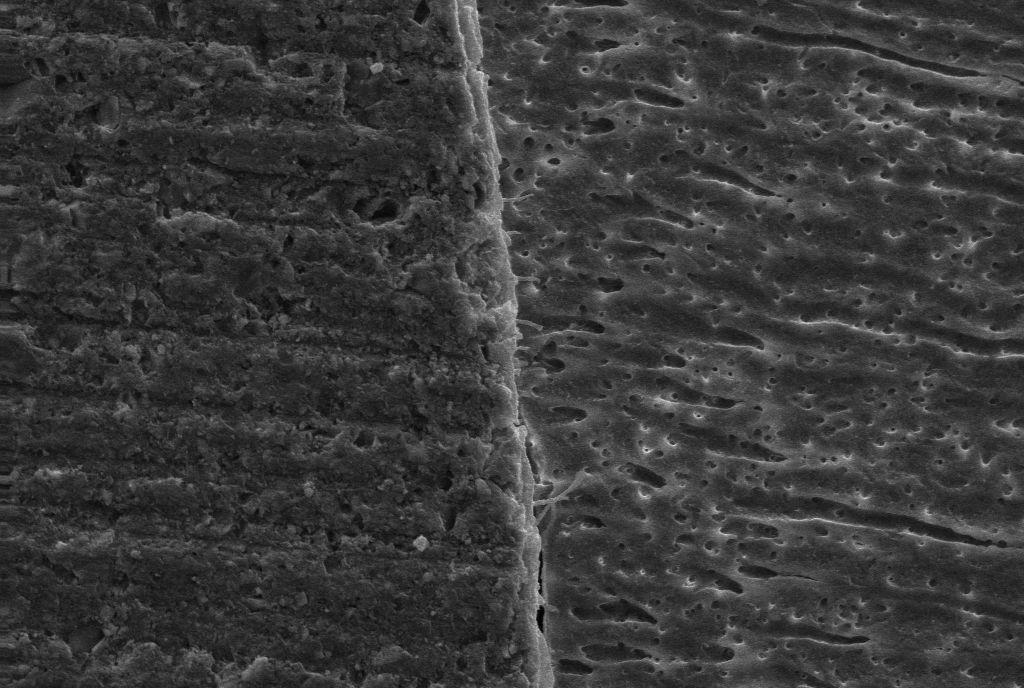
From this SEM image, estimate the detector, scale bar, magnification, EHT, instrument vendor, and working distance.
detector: SE1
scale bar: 10000 nm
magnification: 0.5 K X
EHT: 20 kV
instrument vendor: Zeiss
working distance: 9.5 mm